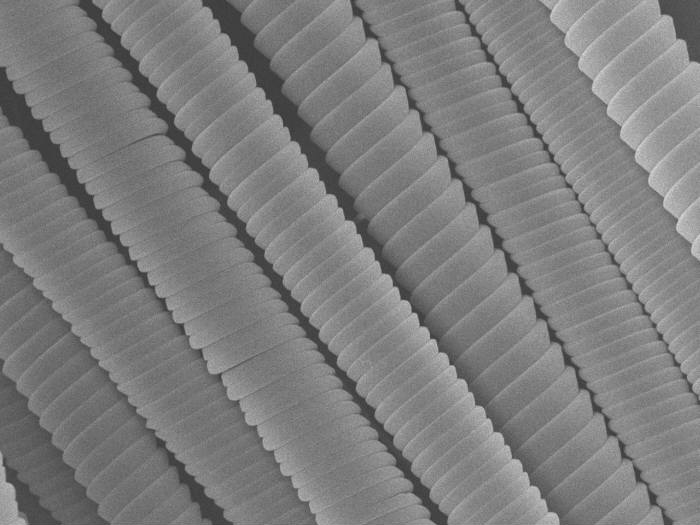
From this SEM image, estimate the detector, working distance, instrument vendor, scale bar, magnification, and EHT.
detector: SEI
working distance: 8.5 mm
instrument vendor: JEOL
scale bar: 1000 nm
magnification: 3 K X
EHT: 15 kV